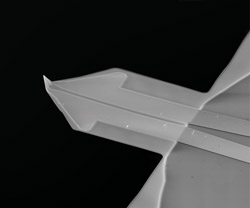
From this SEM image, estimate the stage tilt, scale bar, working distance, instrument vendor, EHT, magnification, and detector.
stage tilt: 57°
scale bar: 100000 nm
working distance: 14.7 mm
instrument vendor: FEI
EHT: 15 kV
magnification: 1.2 K X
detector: ETD